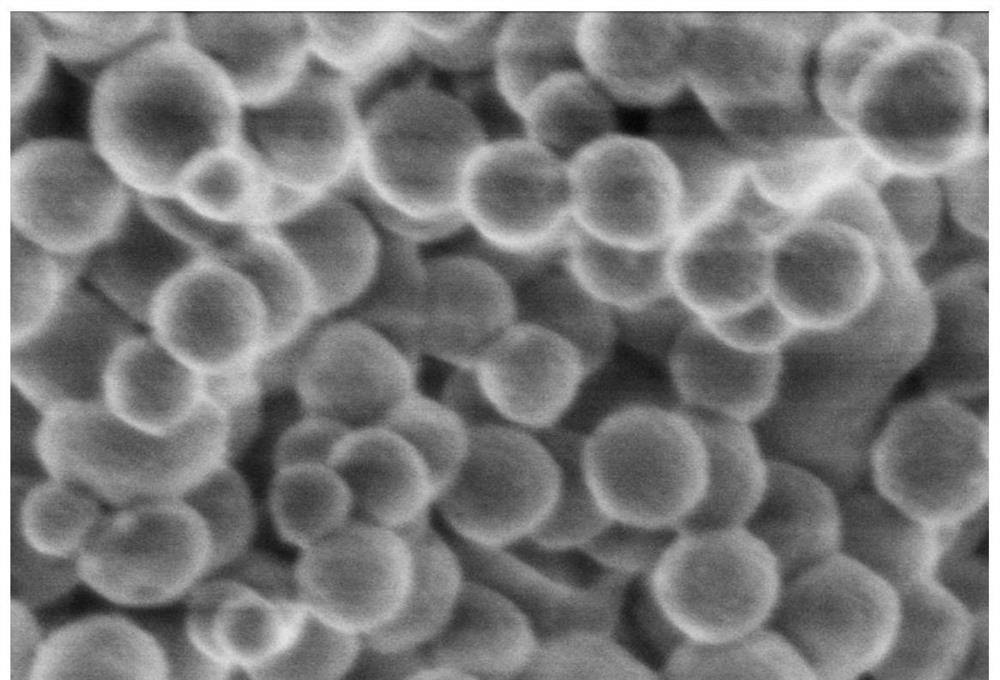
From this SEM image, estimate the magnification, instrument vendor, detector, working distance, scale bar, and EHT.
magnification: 100 K X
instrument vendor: Hitachi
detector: SE(U)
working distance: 6.9 mm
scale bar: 500 nm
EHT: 1 kV